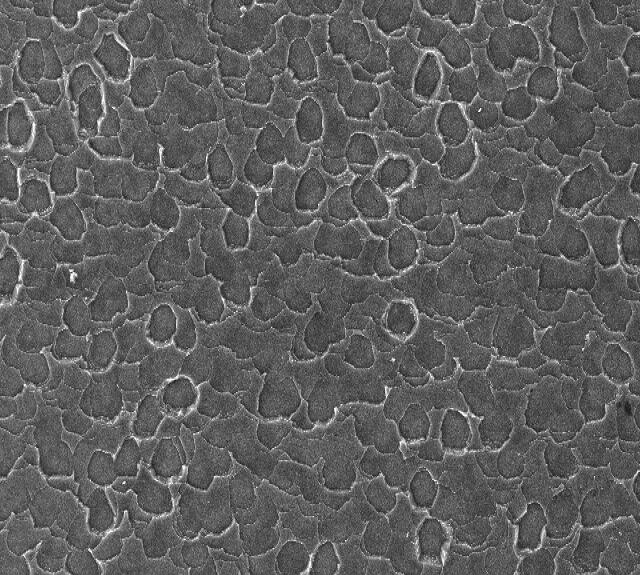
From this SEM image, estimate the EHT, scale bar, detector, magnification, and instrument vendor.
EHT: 30 kV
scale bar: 100000 nm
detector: SEI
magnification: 0.17 K X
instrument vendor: JEOL